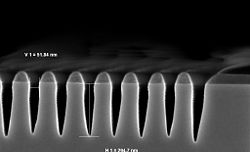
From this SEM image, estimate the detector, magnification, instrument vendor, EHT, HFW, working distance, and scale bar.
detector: InLens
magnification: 200 K X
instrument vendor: Zeiss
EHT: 2 kV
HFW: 1.387 µm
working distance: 3.4 mm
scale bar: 200 nm